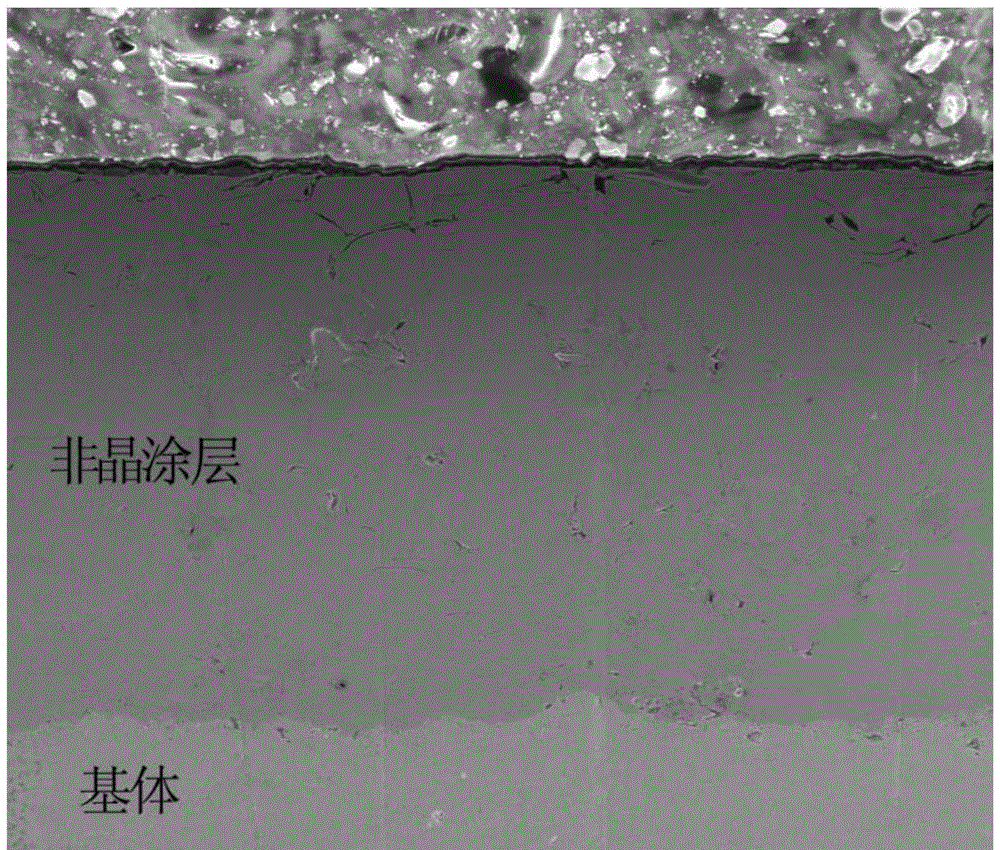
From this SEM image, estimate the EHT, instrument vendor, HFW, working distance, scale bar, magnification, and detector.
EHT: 20 kV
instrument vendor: FEI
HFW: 423 µm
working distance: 10.8 mm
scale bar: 200000 nm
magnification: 0.3 K X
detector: ETD